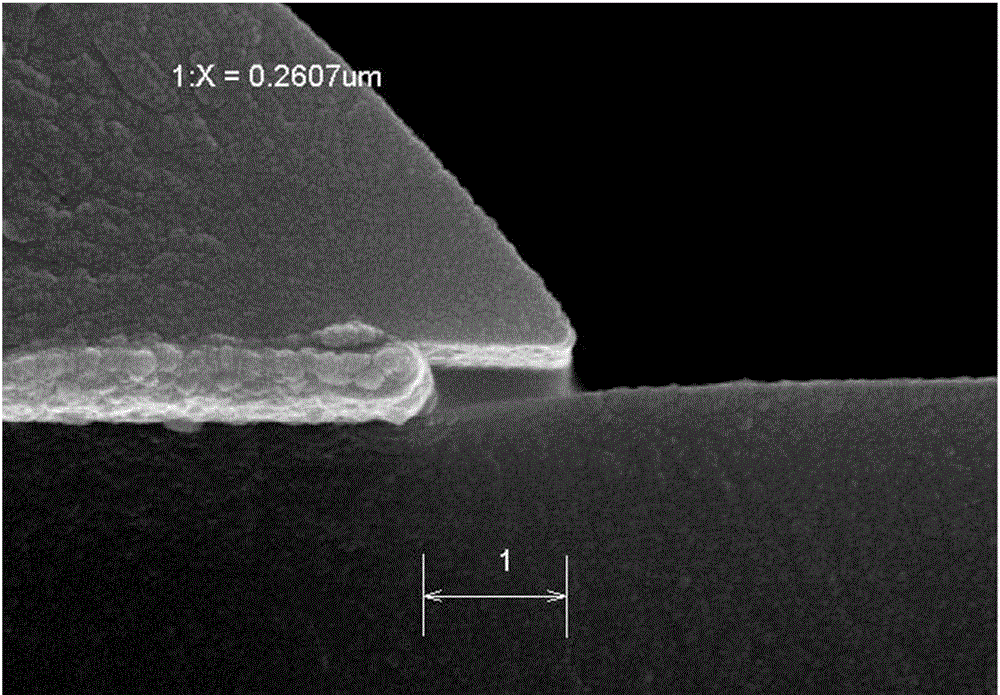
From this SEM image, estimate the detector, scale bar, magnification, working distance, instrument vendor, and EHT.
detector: SE(M)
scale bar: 500 nm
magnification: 70 K X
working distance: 7.5 mm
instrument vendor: Hitachi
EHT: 15 kV